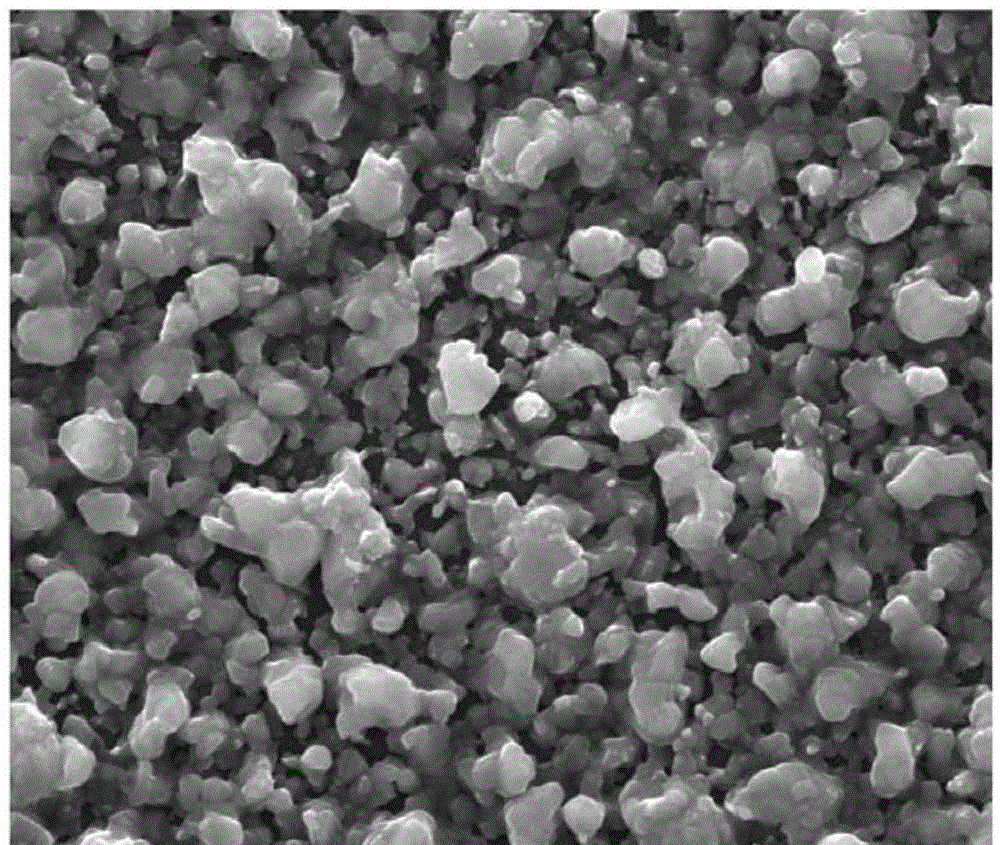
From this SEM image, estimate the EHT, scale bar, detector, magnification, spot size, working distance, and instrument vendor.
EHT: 20 kV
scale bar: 5000 nm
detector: ETD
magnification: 20 K X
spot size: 3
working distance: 10.2 mm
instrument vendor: FEI